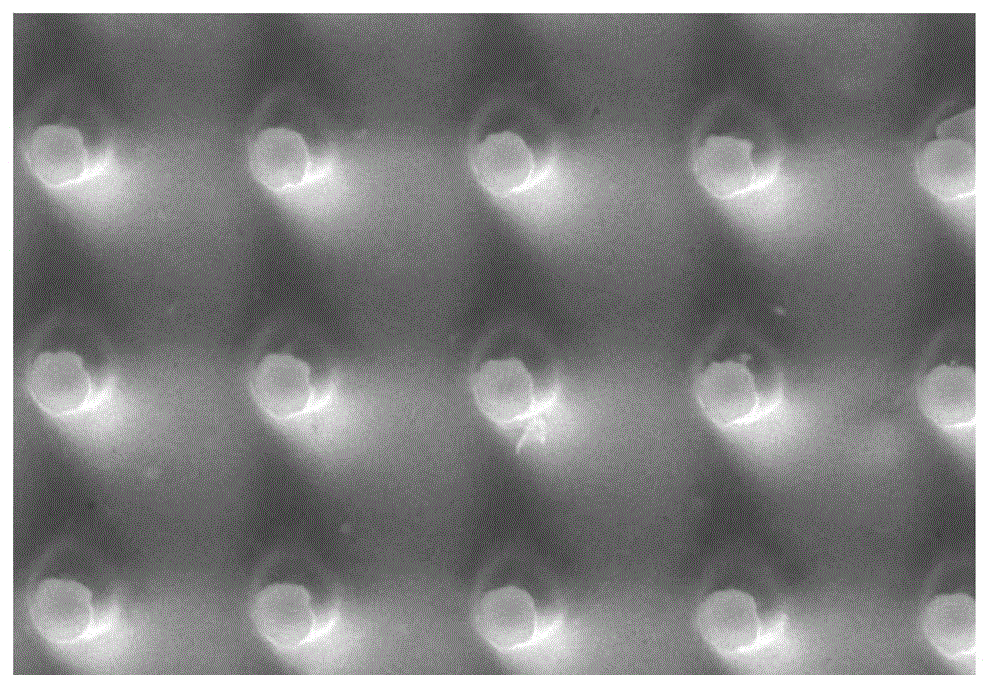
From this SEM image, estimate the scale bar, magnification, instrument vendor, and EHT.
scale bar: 5000 nm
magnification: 3 K X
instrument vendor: JEOL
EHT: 20 kV